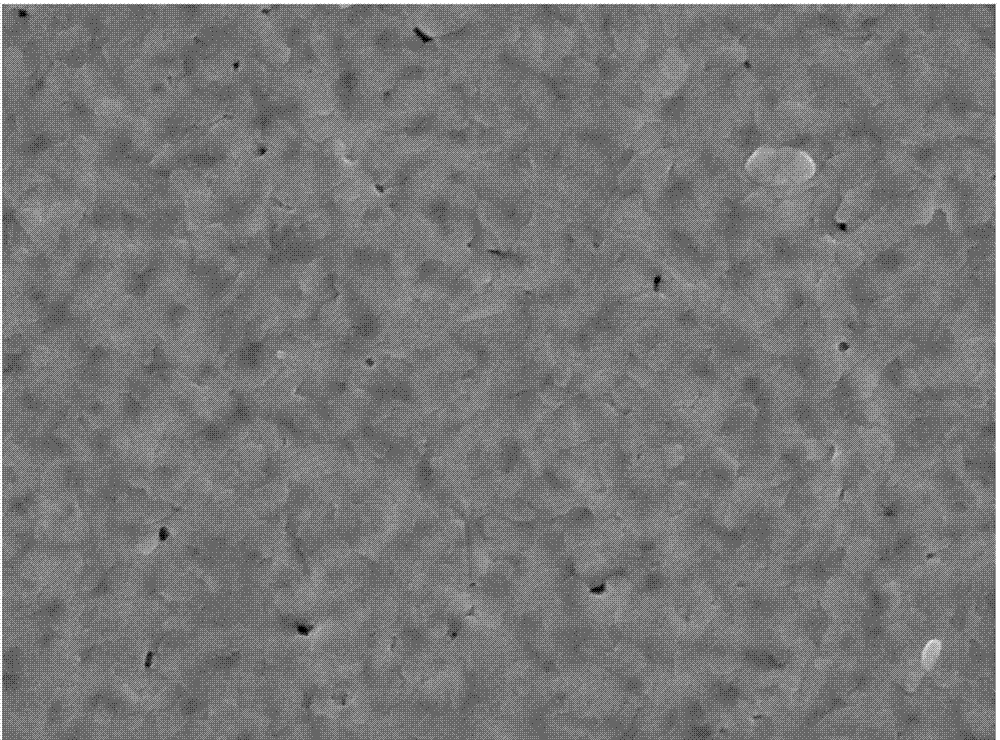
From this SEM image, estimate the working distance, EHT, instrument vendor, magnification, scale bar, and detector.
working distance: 12.8 mm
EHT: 15 kV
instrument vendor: JEOL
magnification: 20 K X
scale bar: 1000 nm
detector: LED